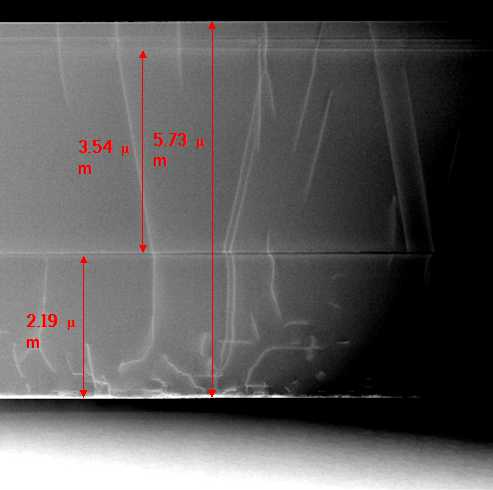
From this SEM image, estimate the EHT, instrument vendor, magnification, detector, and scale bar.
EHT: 200 kV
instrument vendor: JEOL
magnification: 20 K X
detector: STEM BF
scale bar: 2000 nm